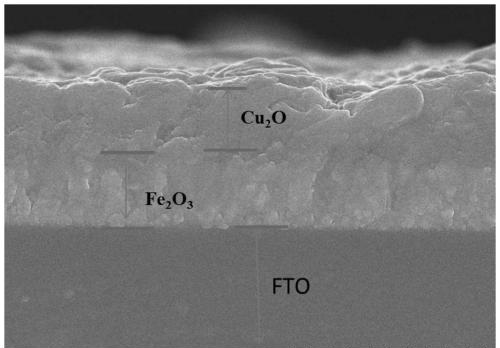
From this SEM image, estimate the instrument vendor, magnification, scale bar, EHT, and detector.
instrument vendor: Hitachi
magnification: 25 K X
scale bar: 2000 nm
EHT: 10 kV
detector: SE(U)